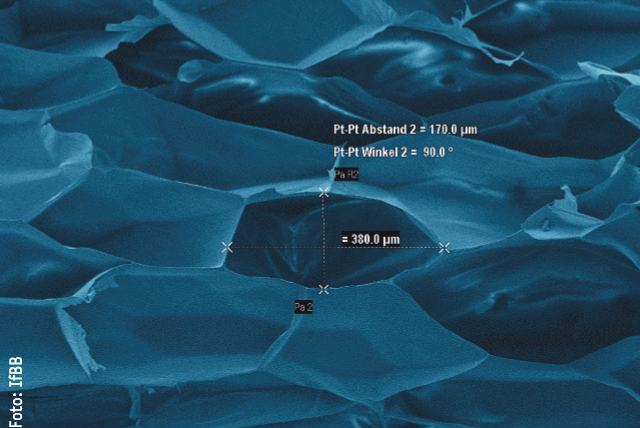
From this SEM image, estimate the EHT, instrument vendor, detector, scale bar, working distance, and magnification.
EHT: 5 kV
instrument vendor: Zeiss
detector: SE1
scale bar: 100000 nm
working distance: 10 mm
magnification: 0.25 K X